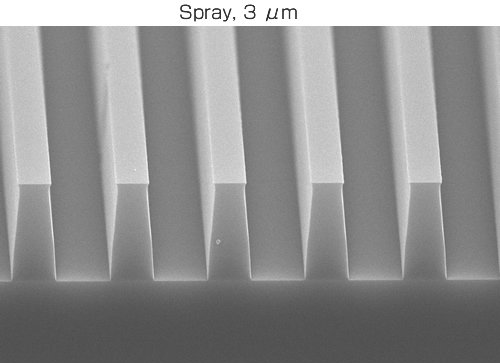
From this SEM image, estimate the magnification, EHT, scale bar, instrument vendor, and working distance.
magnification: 4 K X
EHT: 20 kV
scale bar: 2500 nm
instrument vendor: Hitachi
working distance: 9.5 mm